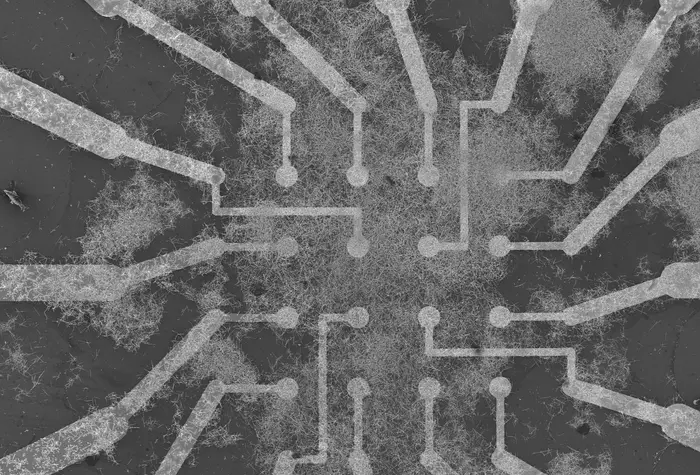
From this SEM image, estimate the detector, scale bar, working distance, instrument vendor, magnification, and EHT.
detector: SEI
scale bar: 200000 nm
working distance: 14 mm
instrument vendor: JEOL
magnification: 0.065 K X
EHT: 10 kV